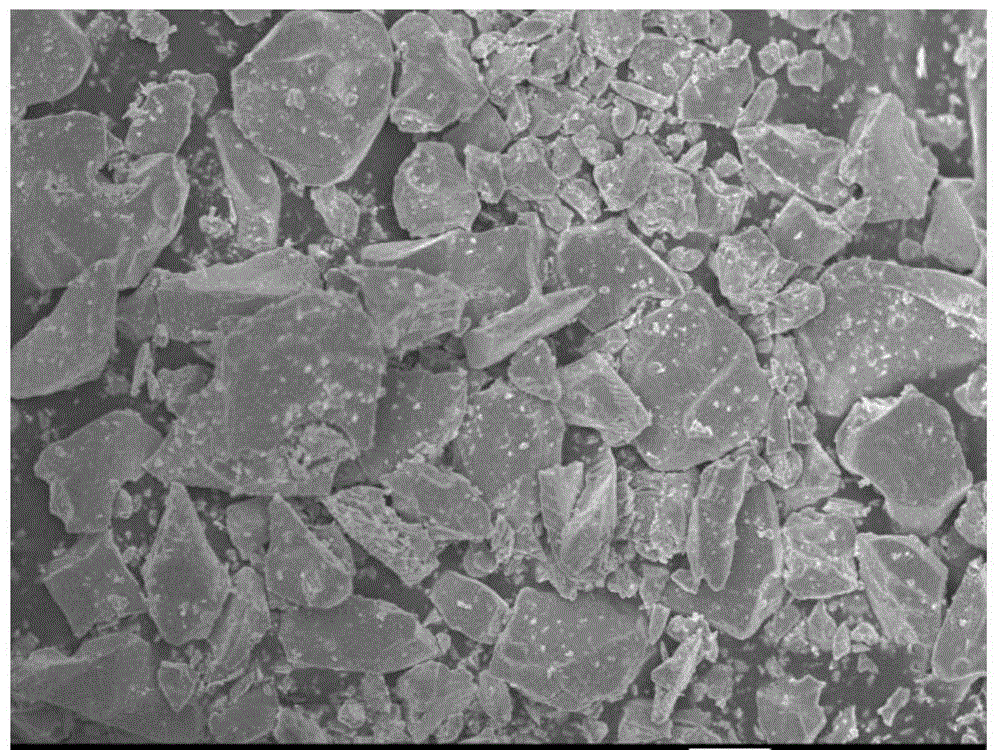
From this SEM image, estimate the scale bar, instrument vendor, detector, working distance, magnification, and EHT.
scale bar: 10000 nm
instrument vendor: JEOL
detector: SEI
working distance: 7.3 mm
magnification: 1 K X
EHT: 10 kV